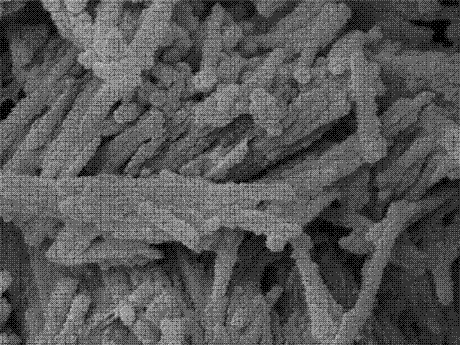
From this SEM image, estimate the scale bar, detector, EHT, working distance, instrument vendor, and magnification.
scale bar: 100 nm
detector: SEI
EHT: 5 kV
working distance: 8 mm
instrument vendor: JEOL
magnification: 40 K X